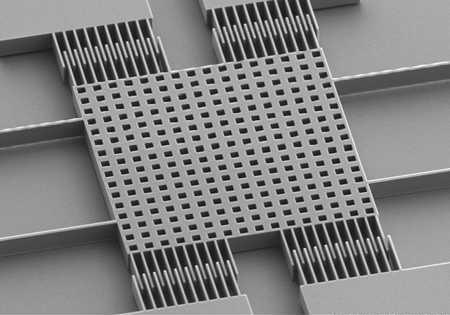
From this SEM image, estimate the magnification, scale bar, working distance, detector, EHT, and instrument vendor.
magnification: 0.3 K X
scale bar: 100000 nm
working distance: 14.5 mm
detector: SE(M)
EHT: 15 kV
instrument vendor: Hitachi